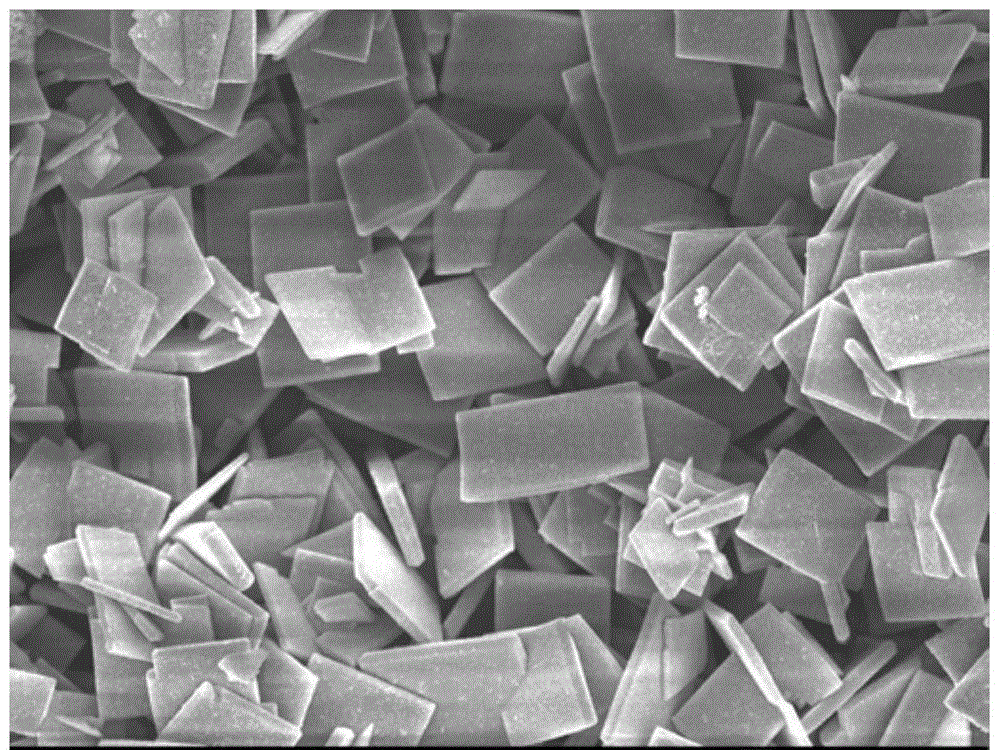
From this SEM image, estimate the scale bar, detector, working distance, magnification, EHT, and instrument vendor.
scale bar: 1000 nm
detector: SEI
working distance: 7.5 mm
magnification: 4 K X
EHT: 8 kV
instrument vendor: JEOL